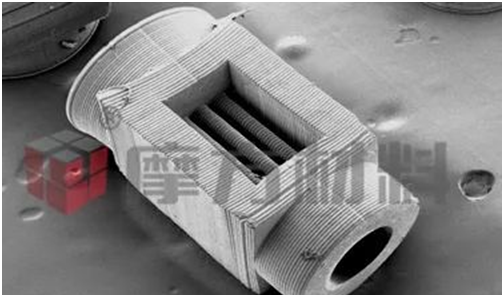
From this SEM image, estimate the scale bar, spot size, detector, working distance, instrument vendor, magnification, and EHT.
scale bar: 500000 nm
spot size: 3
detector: SE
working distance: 9.1 mm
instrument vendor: FEI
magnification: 0.104 K X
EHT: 5 kV